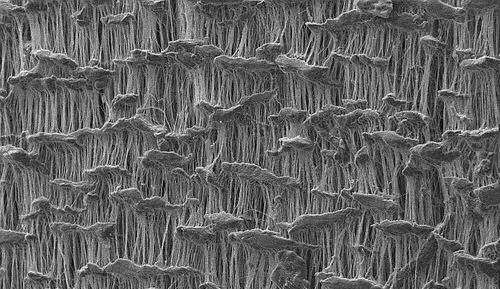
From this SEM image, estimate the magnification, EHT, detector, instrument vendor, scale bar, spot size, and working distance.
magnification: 1 K X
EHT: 10 kV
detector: SE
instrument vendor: FEI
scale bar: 50000 nm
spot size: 3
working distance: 10.5 mm